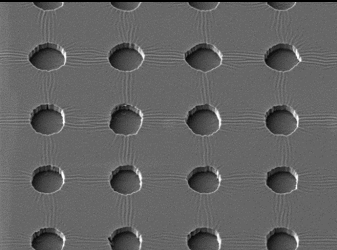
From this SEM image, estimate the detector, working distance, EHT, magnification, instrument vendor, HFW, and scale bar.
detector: SE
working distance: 14.84 mm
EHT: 5 kV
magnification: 3.13 K X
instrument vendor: Tescan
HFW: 111 µm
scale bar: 20000 nm